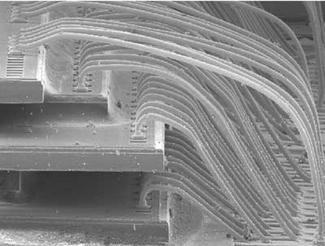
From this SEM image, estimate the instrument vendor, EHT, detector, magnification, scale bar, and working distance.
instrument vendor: JEOL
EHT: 15 kV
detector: LEI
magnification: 0.07 K X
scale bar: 100000 nm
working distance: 26 mm